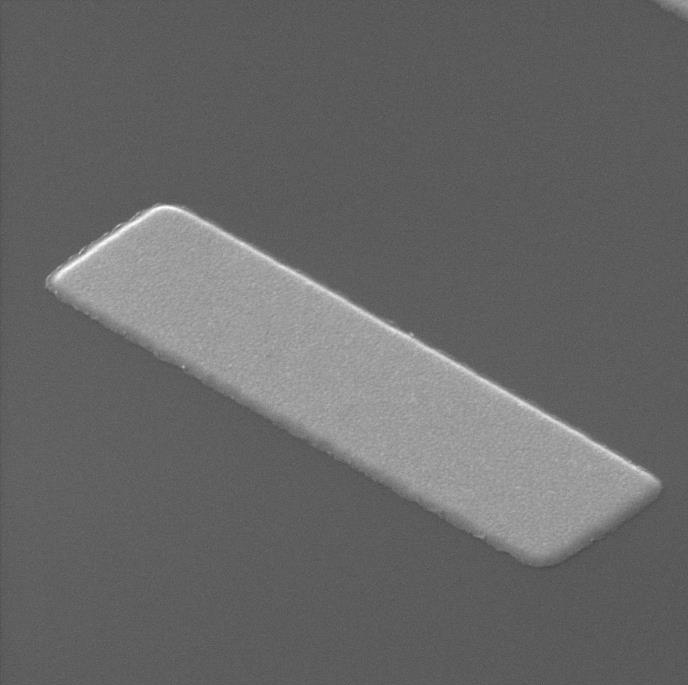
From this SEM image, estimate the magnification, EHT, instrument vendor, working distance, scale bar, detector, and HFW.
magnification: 159 K X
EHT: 30 kV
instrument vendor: Tescan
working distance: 11.55 mm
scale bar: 1000 nm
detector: SE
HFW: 3.47 µm